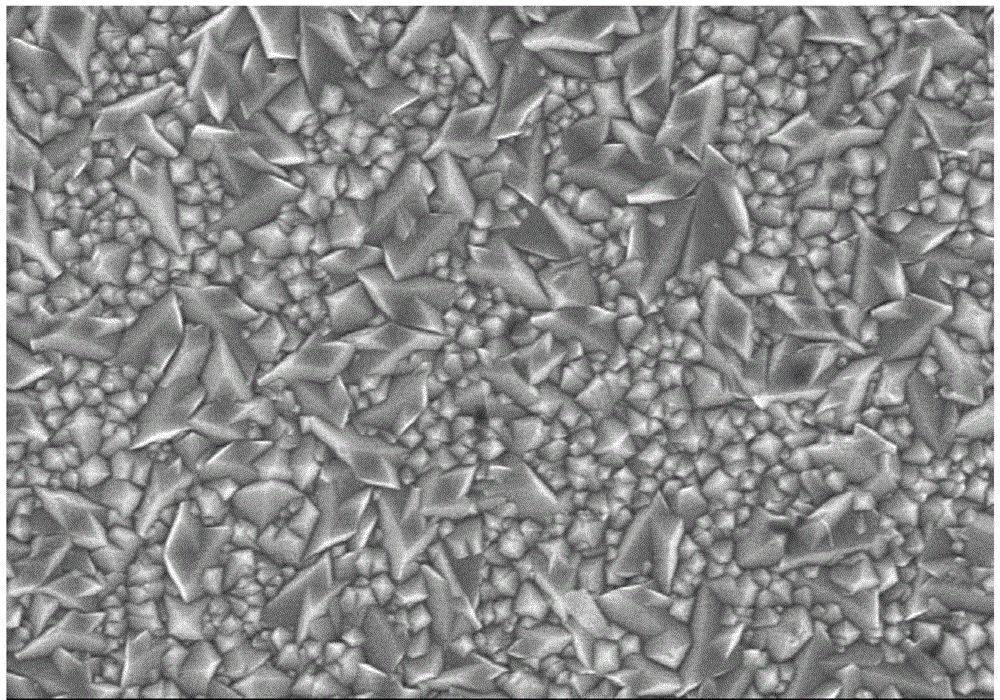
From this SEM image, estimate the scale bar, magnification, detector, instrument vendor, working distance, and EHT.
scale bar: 5000 nm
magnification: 10 K X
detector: SE(U)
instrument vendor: Hitachi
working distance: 10.4 mm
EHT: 8 kV